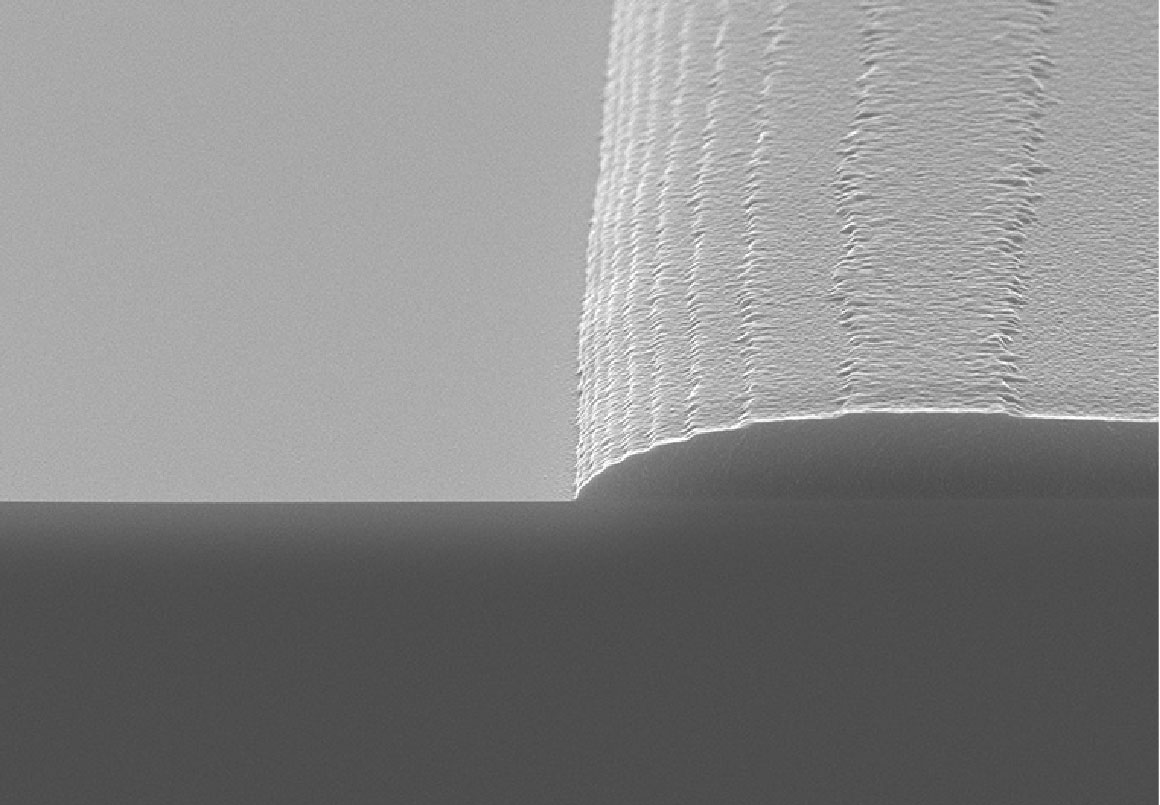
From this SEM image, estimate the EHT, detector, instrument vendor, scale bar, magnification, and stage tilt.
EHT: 10 kV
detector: SE(UL)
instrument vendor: Hitachi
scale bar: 3000 nm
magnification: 10 K X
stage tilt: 80°